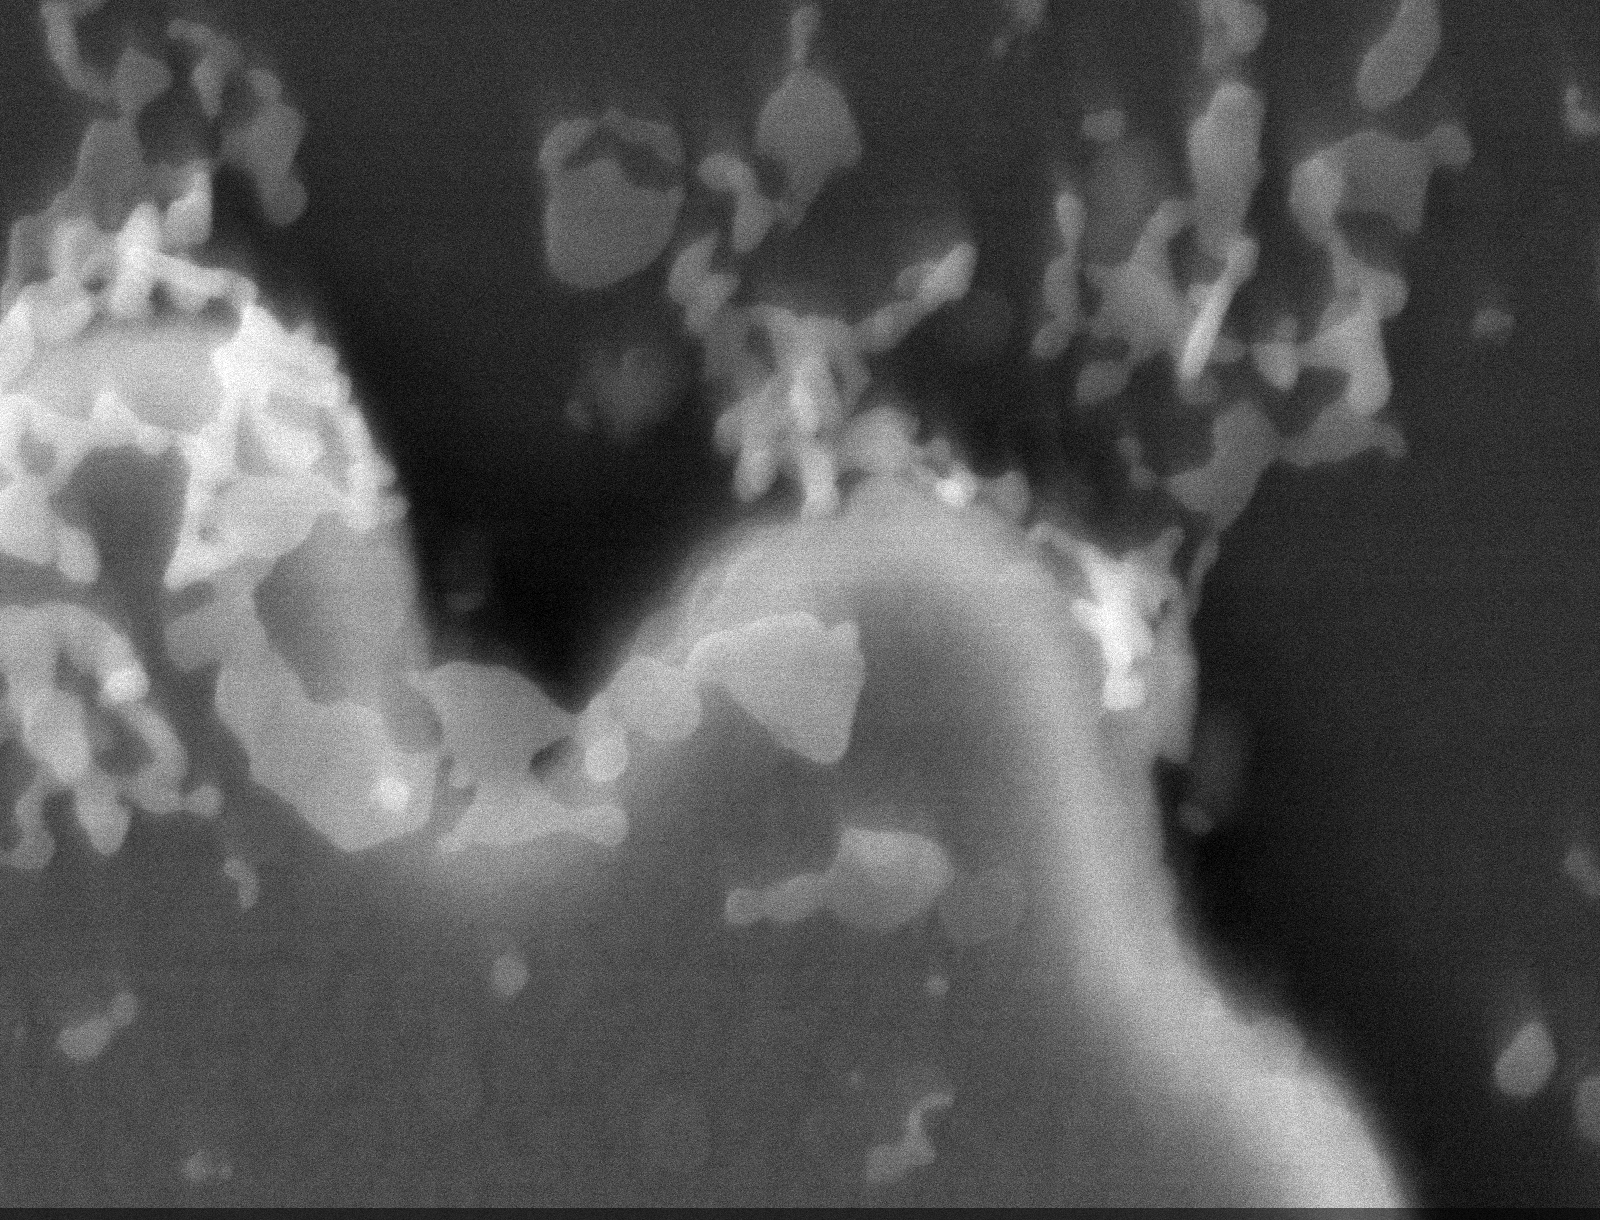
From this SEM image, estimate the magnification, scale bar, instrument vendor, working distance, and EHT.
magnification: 50 K X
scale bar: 400 nm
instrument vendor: Tescan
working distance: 6 mm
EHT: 12 kV